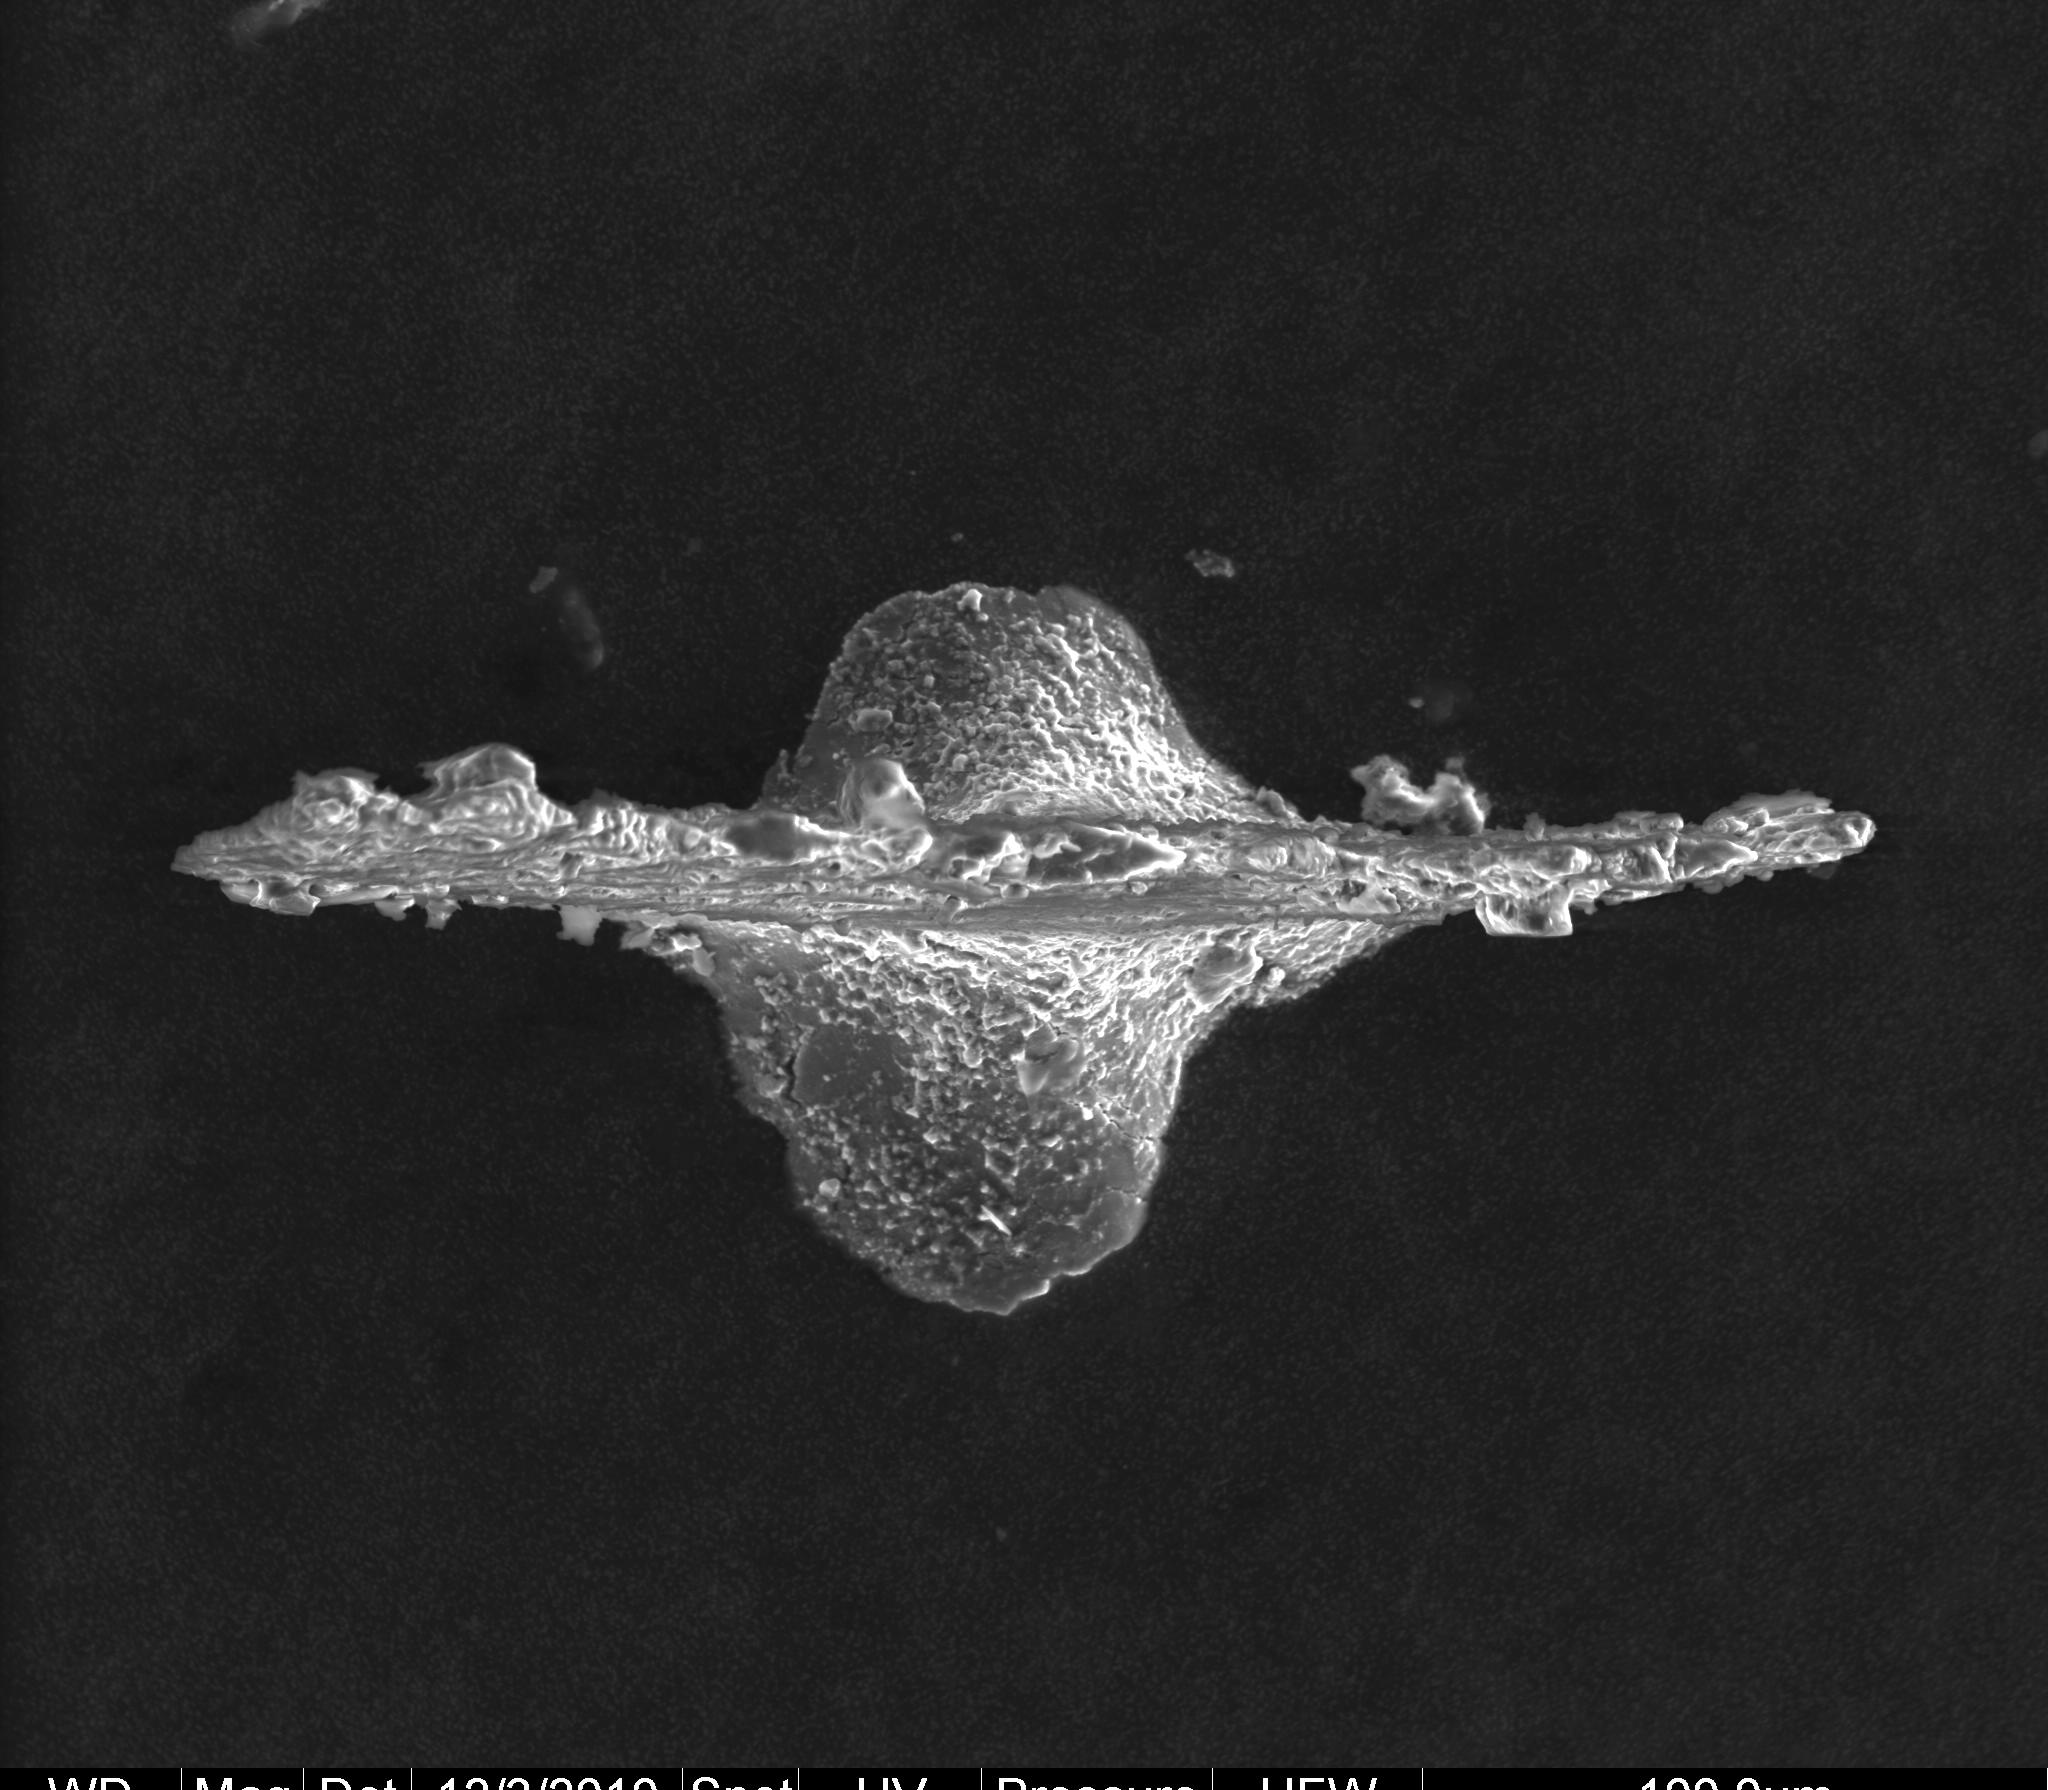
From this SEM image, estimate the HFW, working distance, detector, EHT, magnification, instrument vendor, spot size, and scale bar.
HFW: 450 µm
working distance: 9.6 mm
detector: LFD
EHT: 20 kV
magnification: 0.6 K X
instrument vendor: FEI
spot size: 6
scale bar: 100000 nm